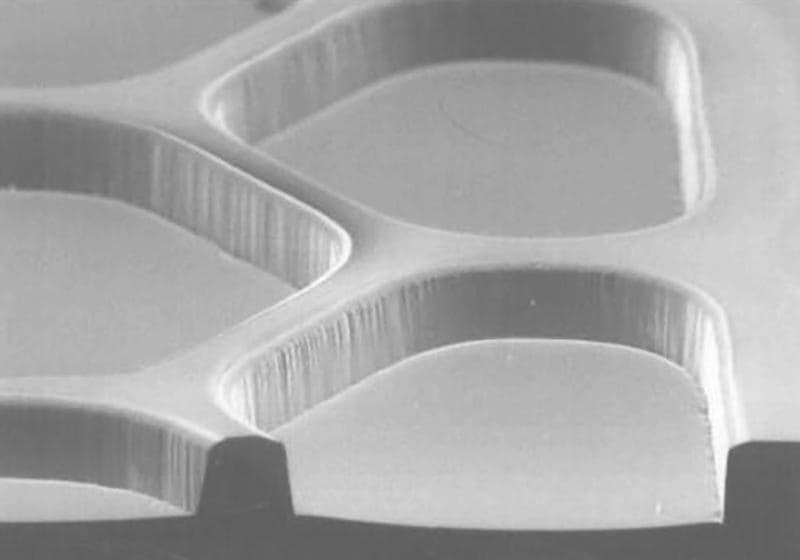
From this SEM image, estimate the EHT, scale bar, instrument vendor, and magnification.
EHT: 15 kV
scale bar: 100000 nm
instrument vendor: JEOL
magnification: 0.15 K X